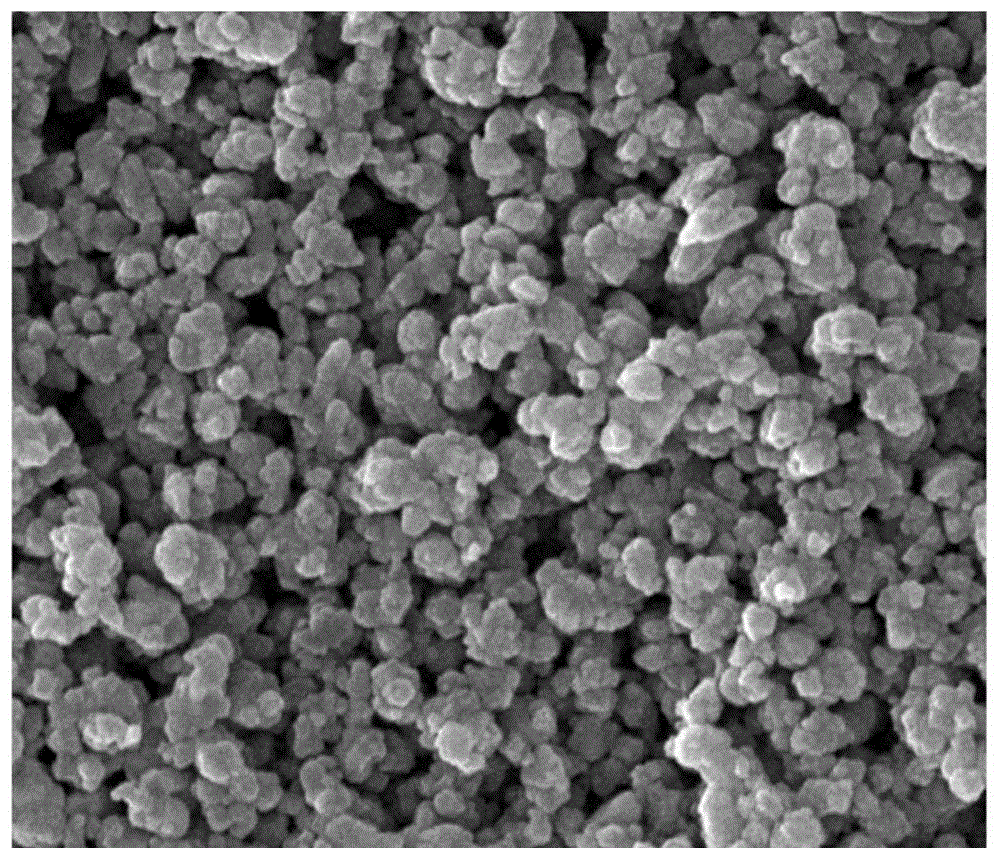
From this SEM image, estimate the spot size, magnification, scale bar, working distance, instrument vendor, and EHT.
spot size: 2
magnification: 48 K X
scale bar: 2000 nm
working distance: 5.6 mm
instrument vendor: FEI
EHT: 25 kV